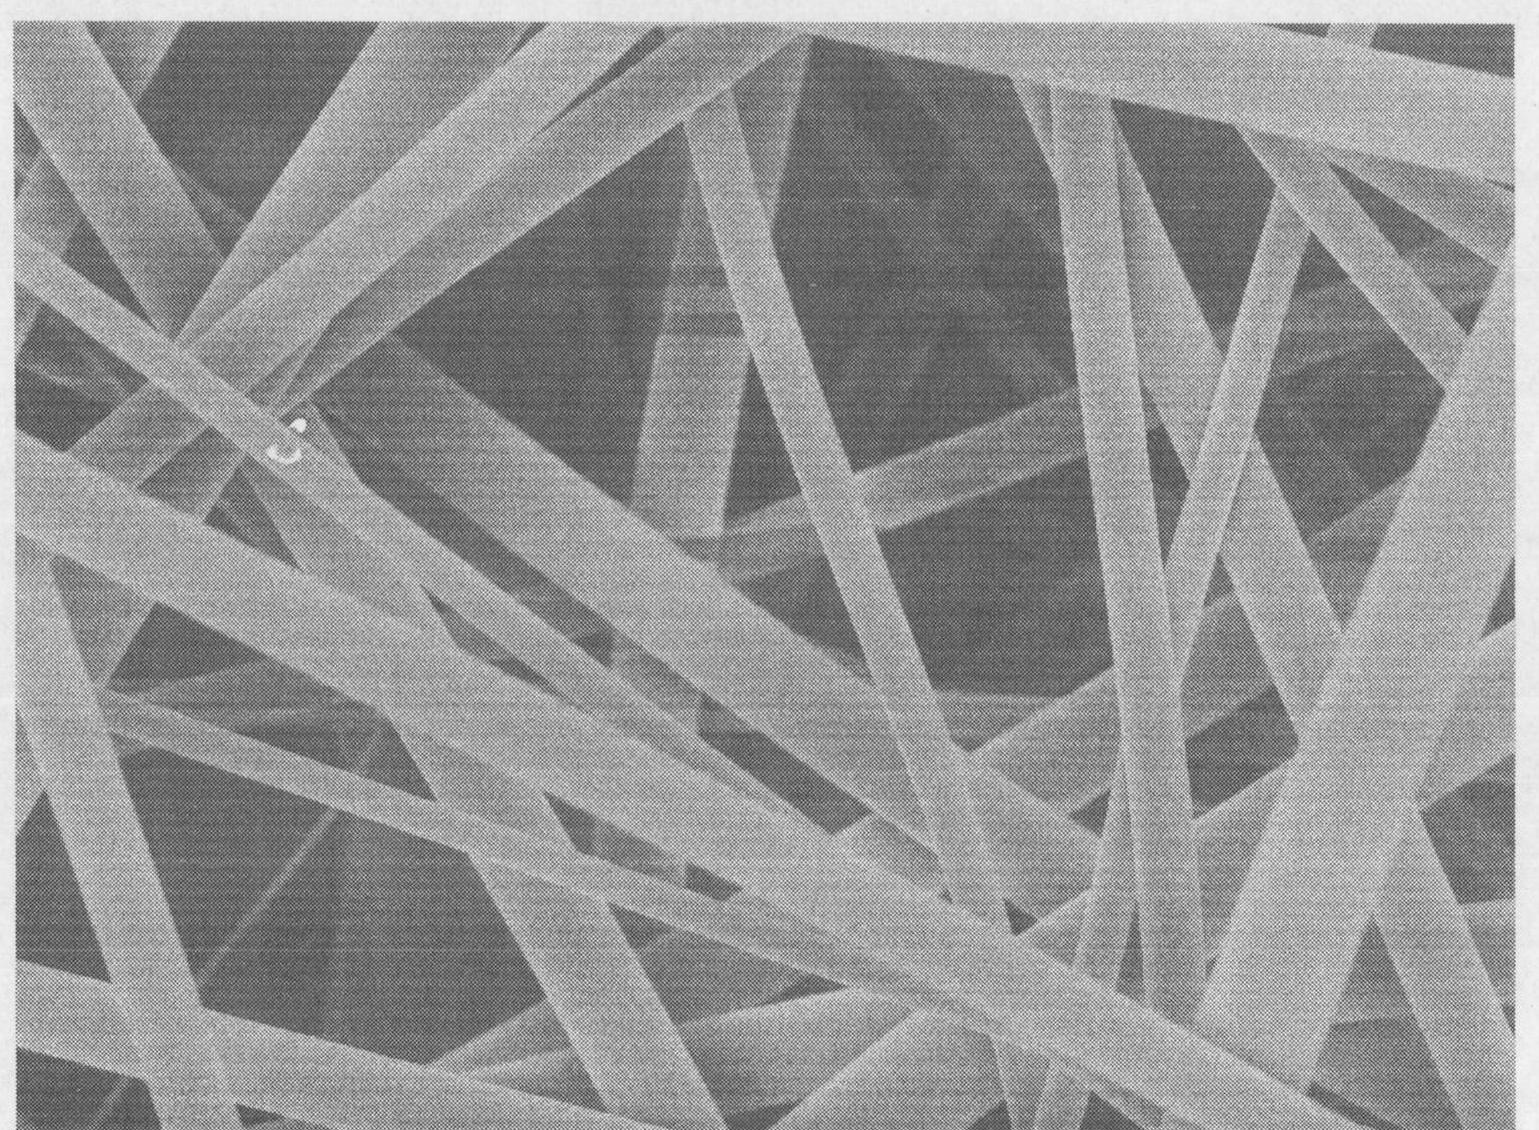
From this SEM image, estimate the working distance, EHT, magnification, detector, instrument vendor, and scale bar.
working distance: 9.7 mm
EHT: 5 kV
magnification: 5 K X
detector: SEI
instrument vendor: JEOL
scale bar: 1000 nm